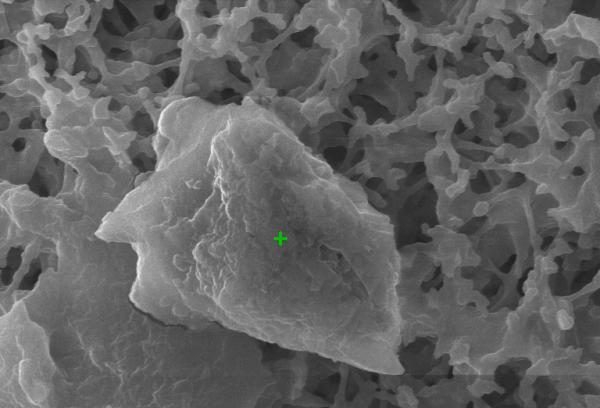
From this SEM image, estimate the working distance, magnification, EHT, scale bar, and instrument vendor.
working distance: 15 mm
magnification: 7.073 K X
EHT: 20 kV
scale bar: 6000 nm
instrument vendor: Tescan